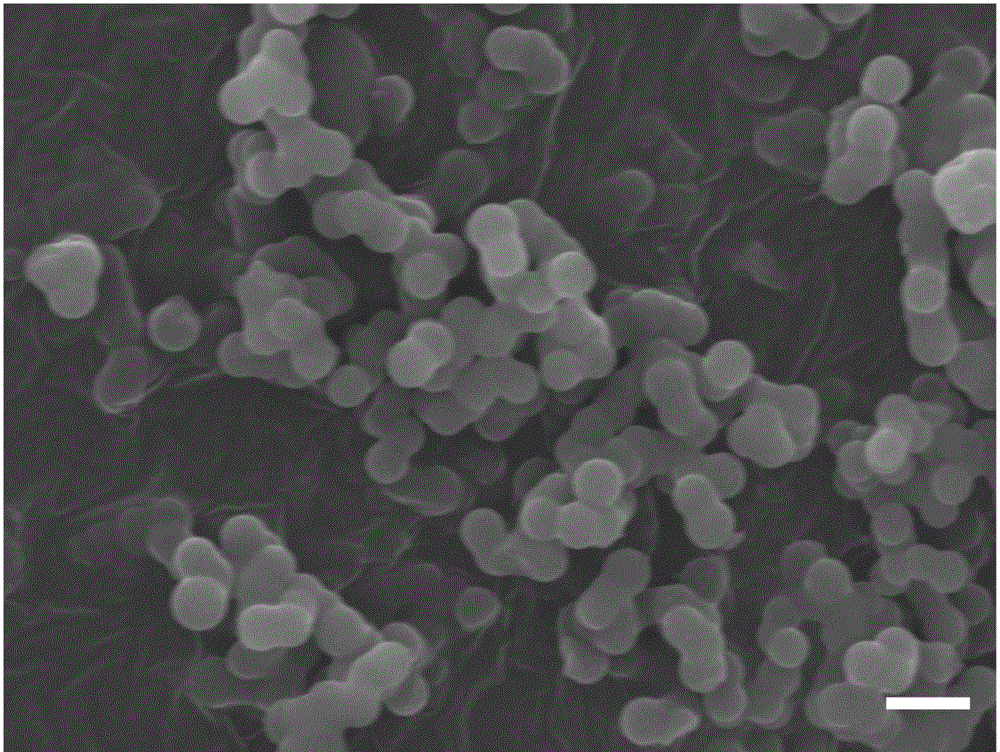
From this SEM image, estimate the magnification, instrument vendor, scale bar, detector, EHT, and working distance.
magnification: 10 K X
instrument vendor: JEOL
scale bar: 1000 nm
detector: SEI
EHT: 15 kV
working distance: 8.5 mm